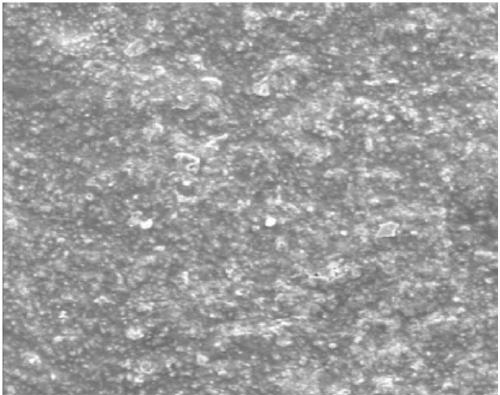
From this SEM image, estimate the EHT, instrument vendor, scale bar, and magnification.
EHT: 10 kV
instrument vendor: JEOL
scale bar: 30000 nm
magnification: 1 K X